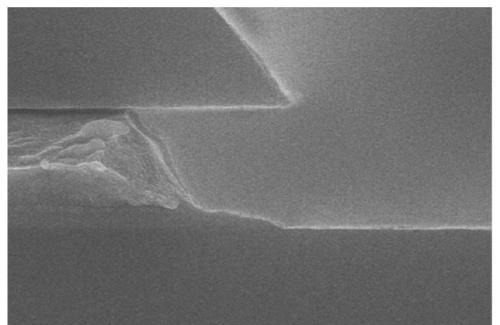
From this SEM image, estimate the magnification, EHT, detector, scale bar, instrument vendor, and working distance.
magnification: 50 K X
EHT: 10 kV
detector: SE(U)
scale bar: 1000 nm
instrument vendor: Hitachi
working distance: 9.6 mm